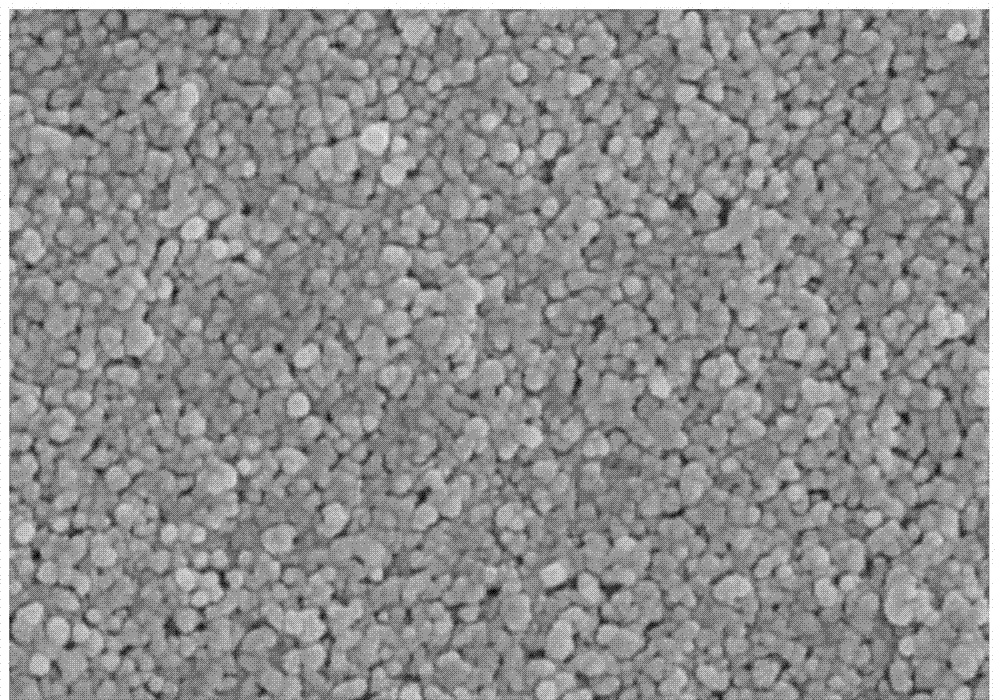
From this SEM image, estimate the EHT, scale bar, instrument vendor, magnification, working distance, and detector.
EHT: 5 kV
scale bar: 1000 nm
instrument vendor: Hitachi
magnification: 50 K X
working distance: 9 mm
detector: SE(M)